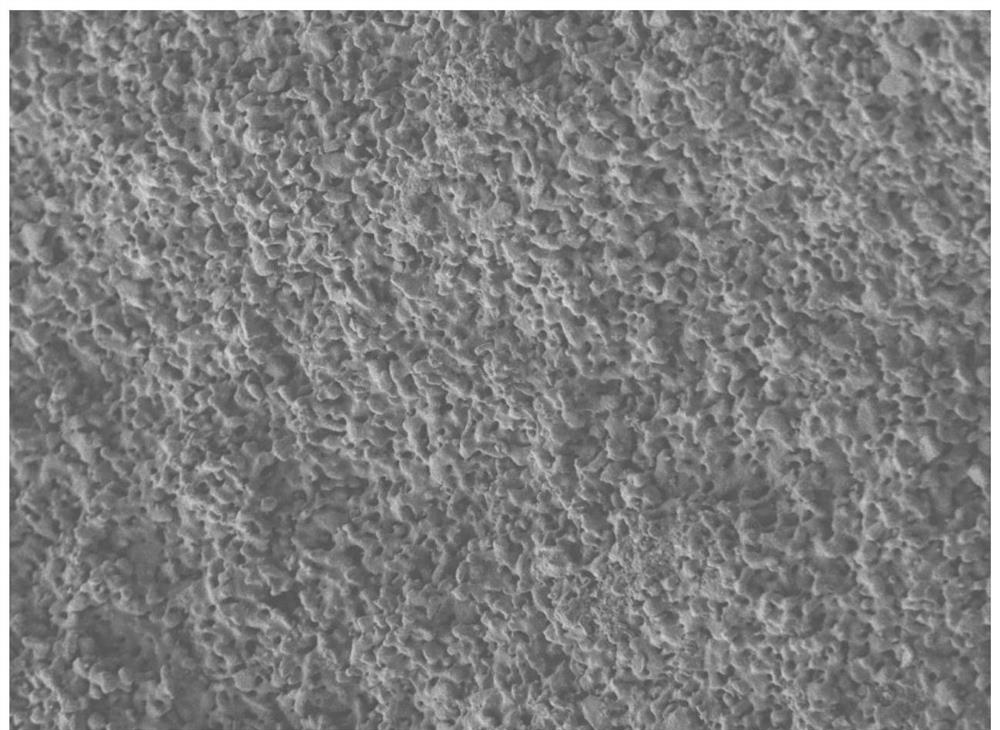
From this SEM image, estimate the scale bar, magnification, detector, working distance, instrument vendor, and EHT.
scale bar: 100 nm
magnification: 40 K X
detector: SEI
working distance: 8.2 mm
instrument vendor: JEOL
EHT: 5 kV